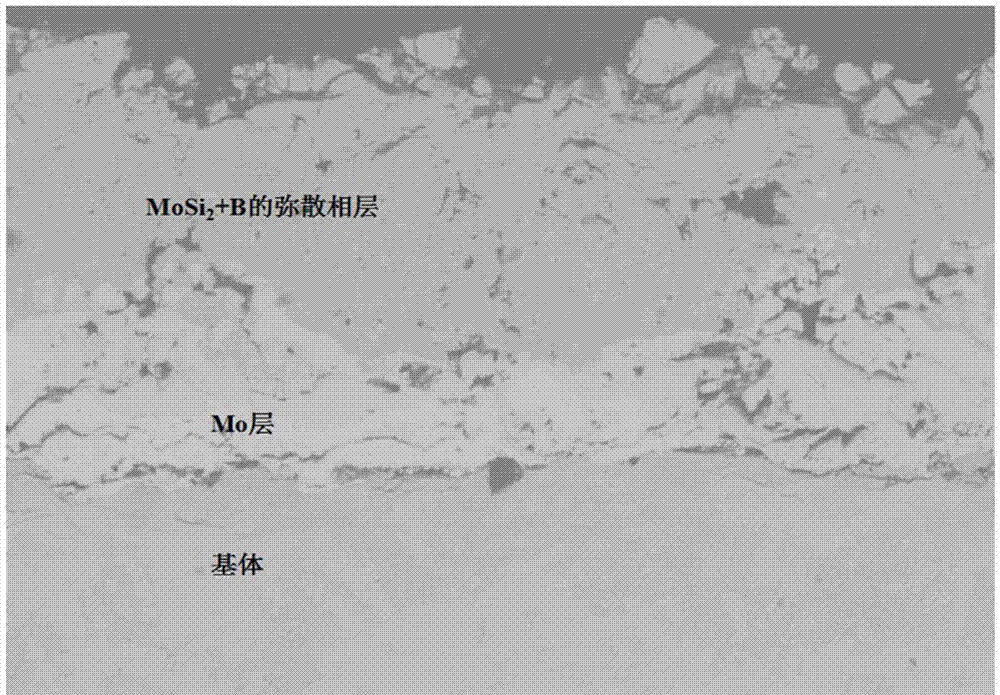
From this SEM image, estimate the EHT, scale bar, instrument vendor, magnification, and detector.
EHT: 20 kV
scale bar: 10000 nm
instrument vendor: JEOL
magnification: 0.3 K X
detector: COMPO